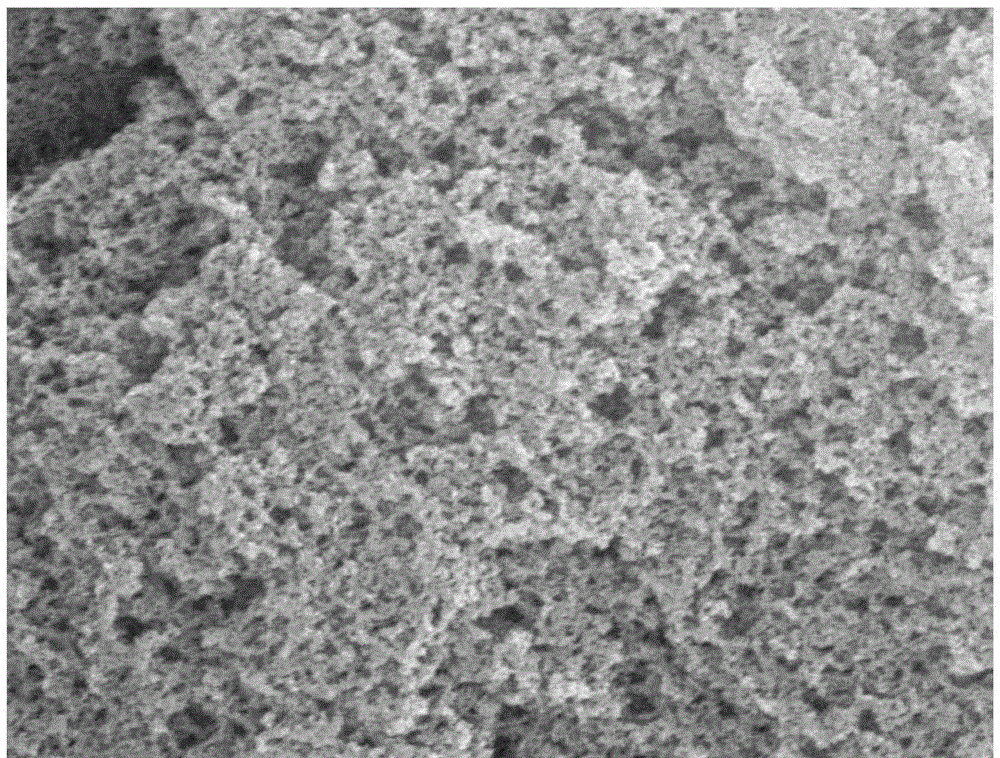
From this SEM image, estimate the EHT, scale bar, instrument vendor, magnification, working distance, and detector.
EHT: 20 kV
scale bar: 1000 nm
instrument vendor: FEI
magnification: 50 K X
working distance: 7.3 mm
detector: SE